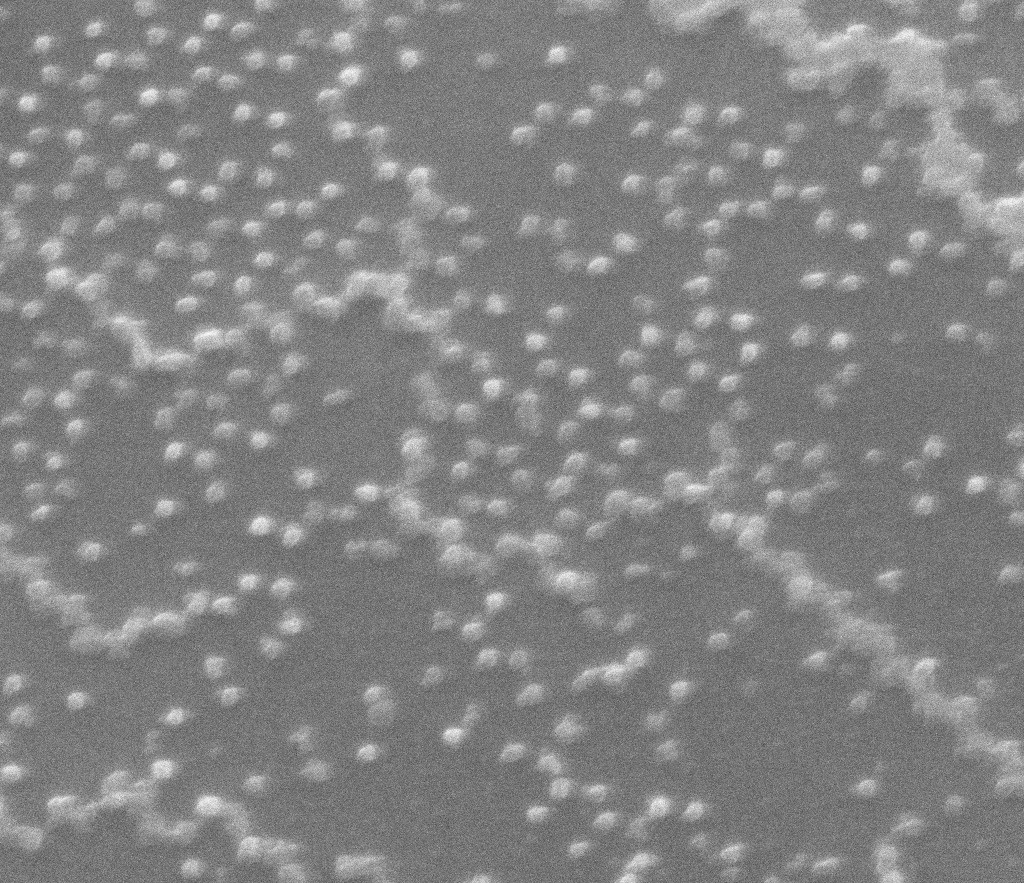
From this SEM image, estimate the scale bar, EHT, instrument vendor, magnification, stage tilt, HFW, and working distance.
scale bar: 3000 nm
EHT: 25 kV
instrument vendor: FEI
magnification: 30.234 K X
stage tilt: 0°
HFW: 8.94 µm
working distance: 12 mm